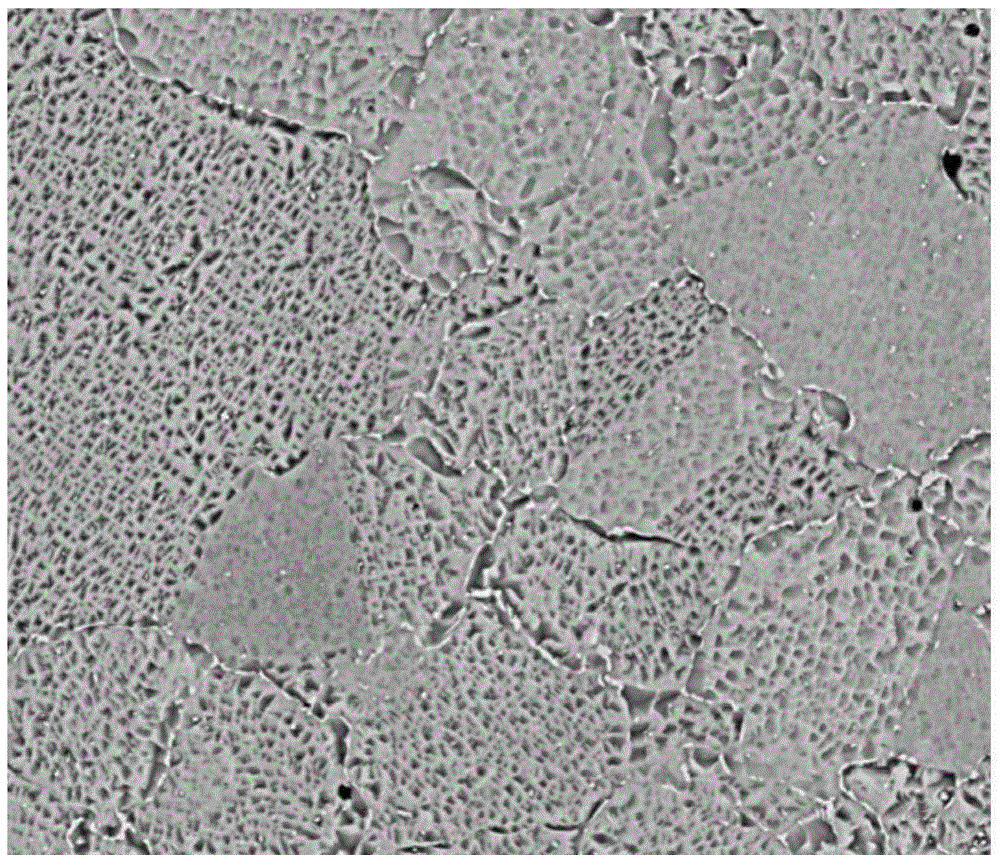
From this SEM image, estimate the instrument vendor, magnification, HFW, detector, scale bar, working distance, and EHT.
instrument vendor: FEI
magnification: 5 K X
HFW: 59.7 µm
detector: BSED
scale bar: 20000 nm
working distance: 11.4 mm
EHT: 20 kV